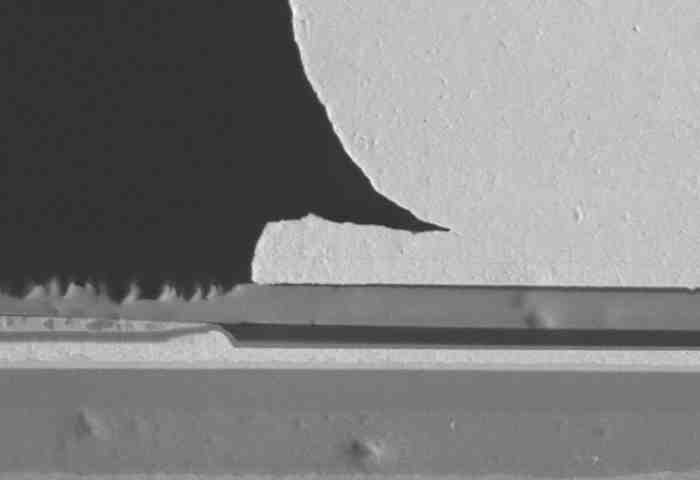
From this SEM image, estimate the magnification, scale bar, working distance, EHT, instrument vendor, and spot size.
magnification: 2 K X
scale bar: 10000 nm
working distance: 17.8 mm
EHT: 20 kV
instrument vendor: other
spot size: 4